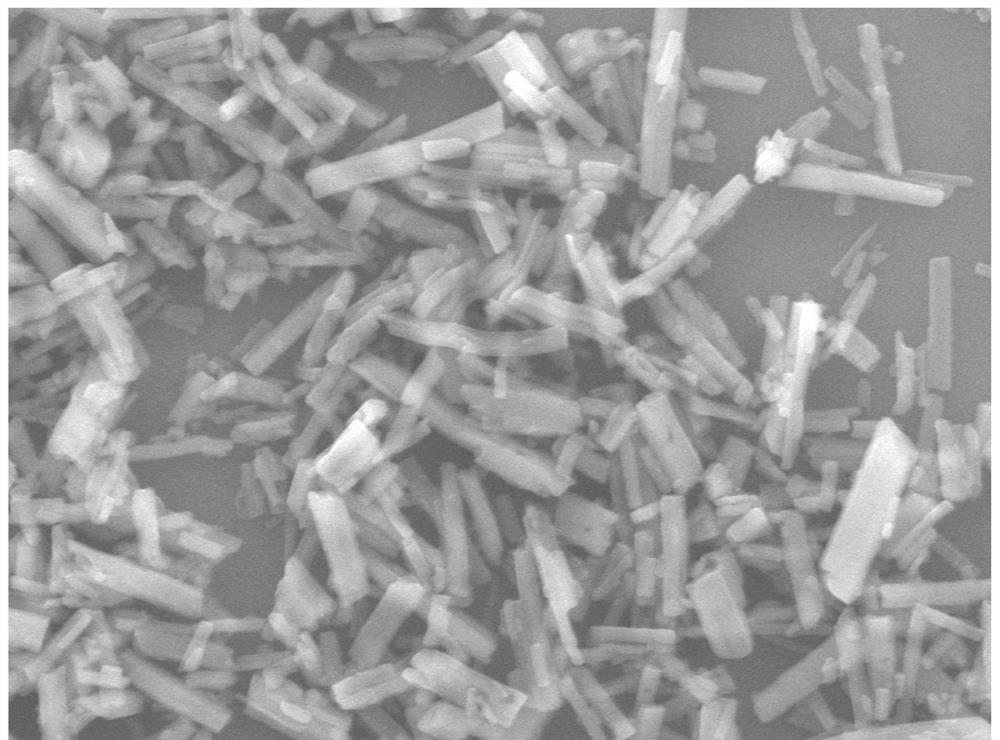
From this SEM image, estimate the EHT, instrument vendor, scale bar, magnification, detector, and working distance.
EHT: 10 kV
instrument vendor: JEOL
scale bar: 1000 nm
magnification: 10 K X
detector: LED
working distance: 7.9 mm